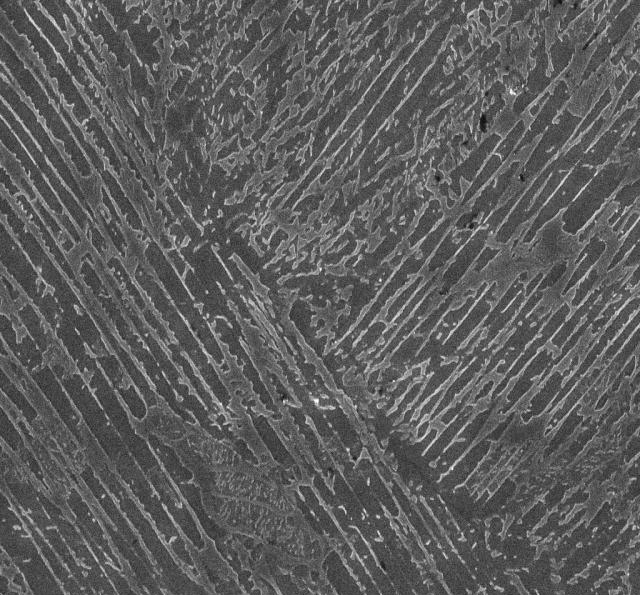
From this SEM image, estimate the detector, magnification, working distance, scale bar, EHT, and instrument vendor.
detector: InLens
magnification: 10 K X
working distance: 8.1 mm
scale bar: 2000 nm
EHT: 15 kV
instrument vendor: Zeiss